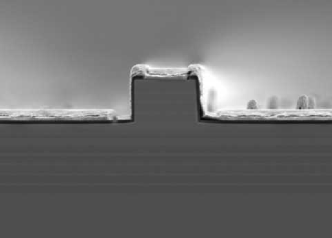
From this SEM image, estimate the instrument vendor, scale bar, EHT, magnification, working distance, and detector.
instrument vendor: JEOL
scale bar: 1000 nm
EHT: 5 kV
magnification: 7 K X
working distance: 6.2 mm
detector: LED